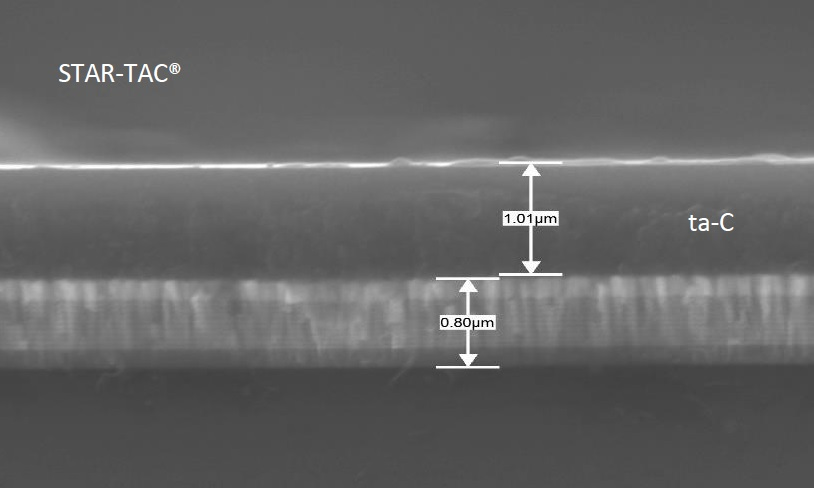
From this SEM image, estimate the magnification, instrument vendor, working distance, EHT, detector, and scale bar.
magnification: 20 K X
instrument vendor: JEOL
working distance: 10 mm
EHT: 15 kV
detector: SEI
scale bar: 1000 nm